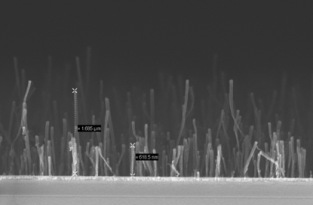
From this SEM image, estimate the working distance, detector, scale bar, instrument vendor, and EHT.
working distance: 6 mm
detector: InLens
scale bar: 200 nm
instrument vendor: Zeiss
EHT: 10 kV